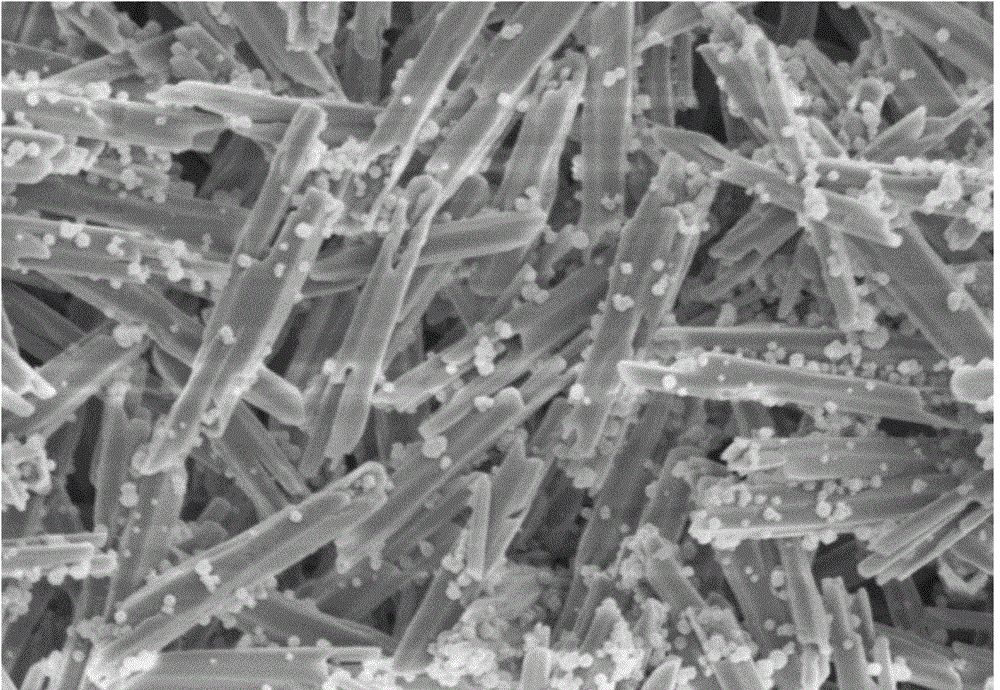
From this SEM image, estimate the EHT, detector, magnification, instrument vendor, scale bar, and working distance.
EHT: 5 kV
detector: SE(M)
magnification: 8 K X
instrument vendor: Hitachi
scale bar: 5000 nm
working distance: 10.2 mm